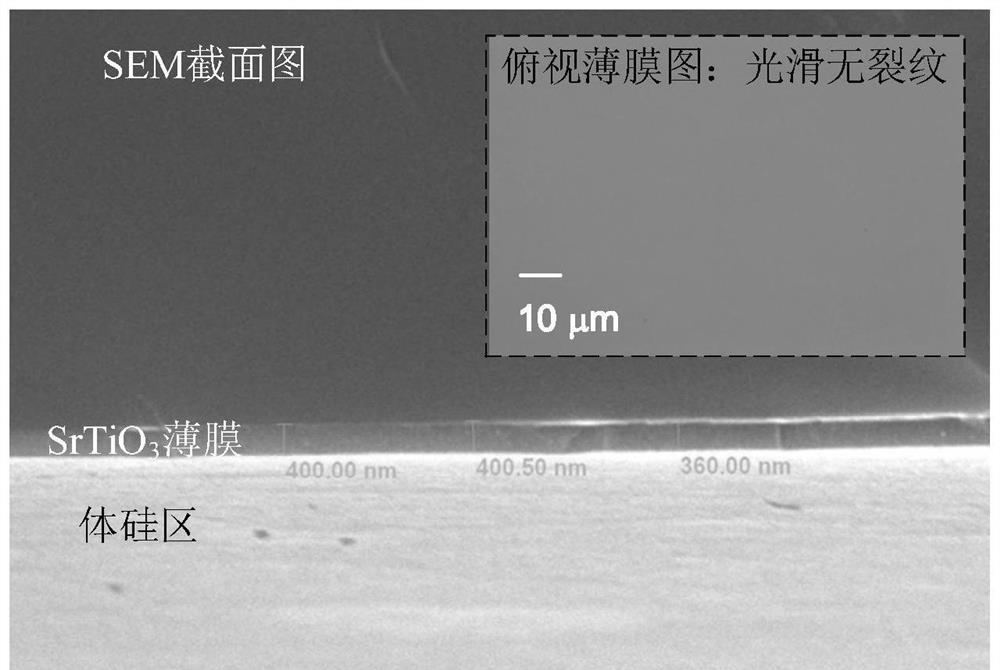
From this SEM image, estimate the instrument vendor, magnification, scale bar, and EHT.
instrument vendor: JEOL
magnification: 10 K X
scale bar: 1000 nm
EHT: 20 kV